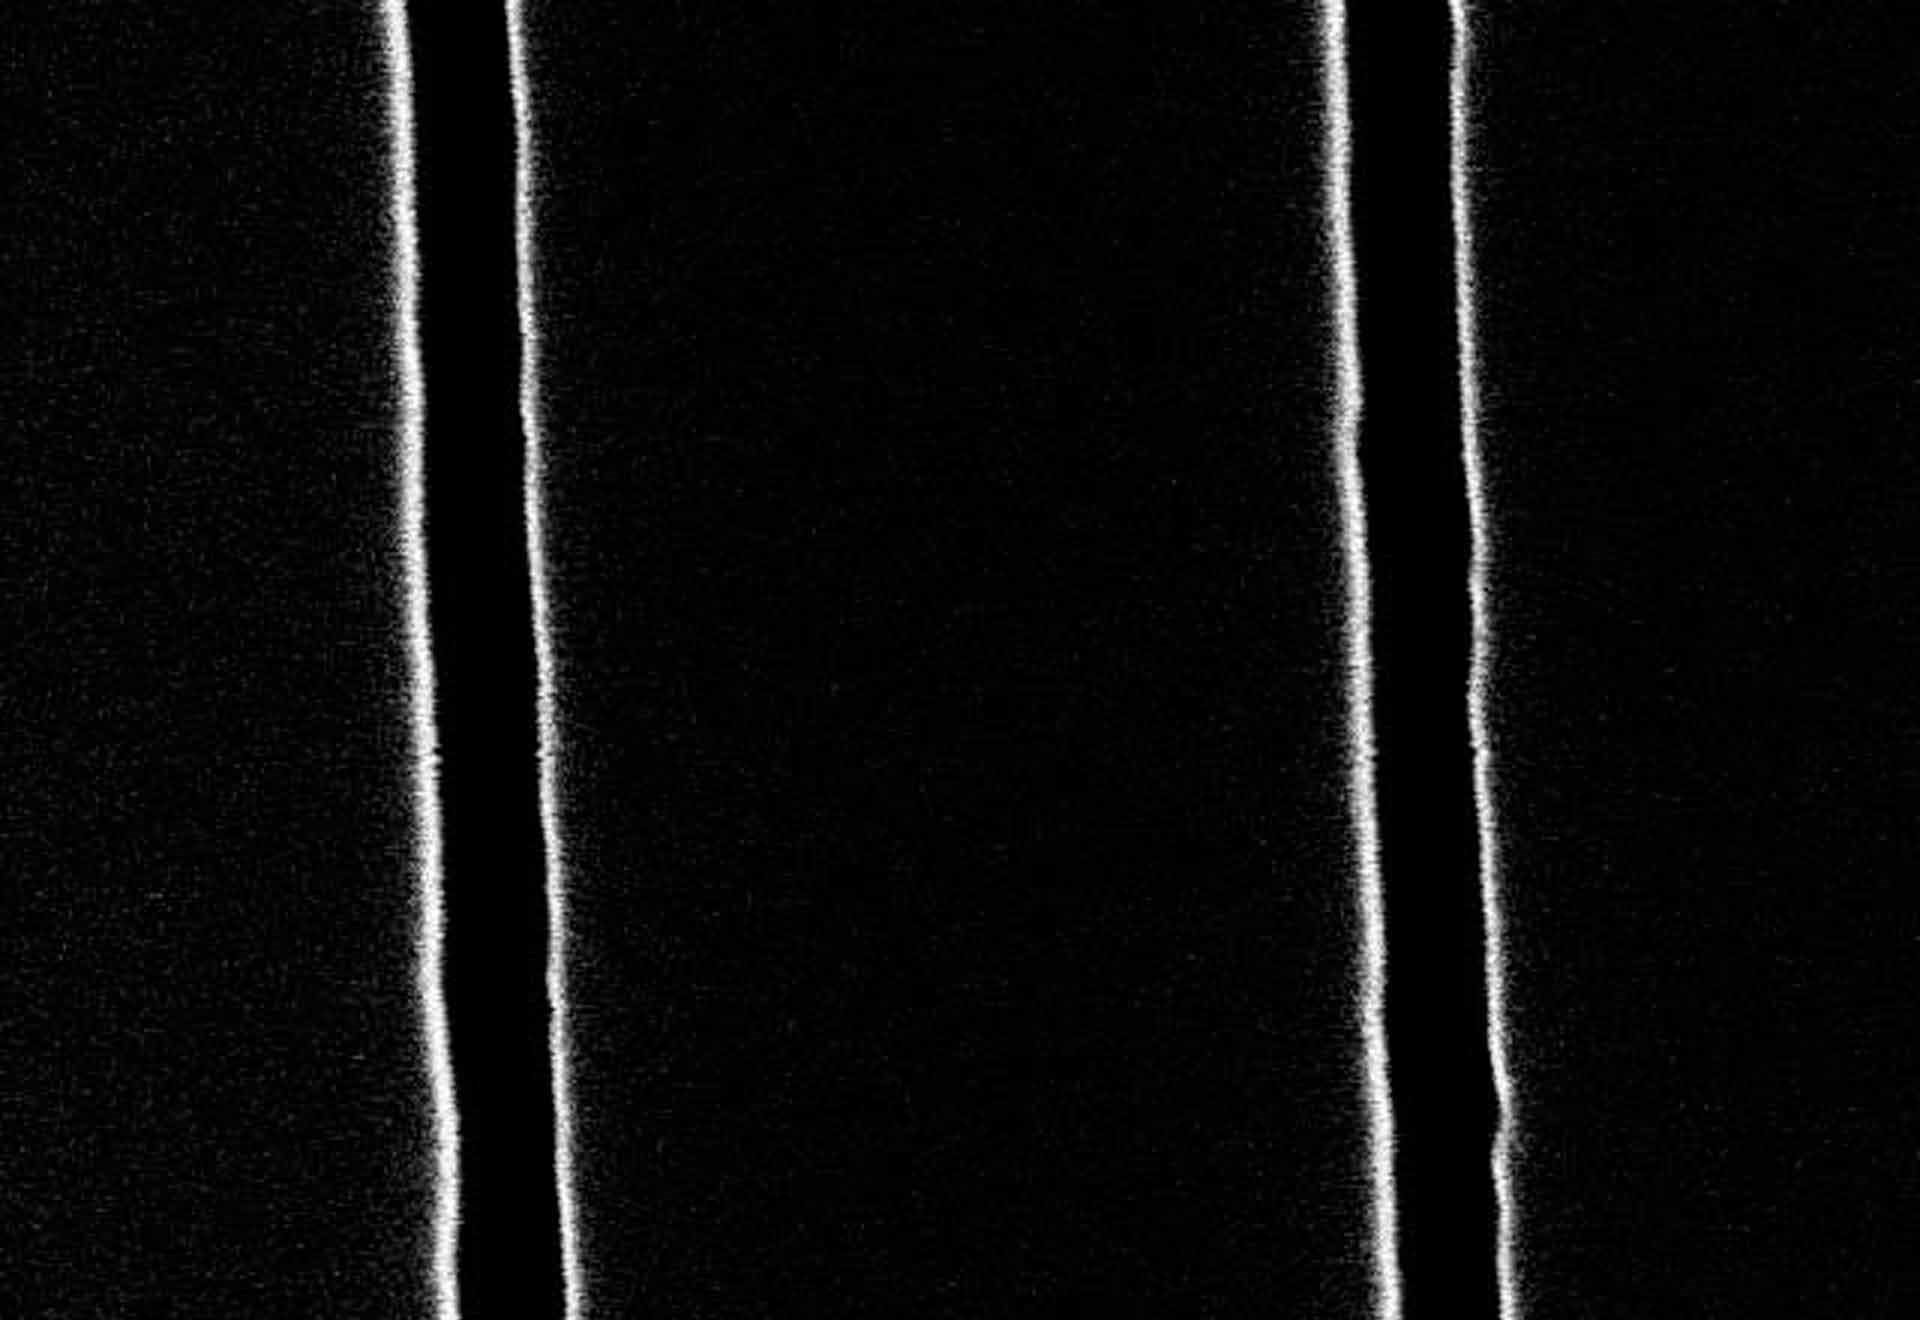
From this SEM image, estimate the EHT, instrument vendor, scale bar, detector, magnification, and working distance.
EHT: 15 kV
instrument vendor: Hitachi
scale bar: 400 nm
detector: SE(M)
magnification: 120 K X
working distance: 19.5 mm